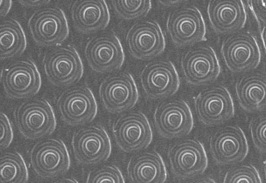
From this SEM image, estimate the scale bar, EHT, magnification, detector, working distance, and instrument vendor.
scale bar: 1000 nm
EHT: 3 kV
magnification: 3 K X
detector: SEI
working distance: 15 mm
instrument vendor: JEOL